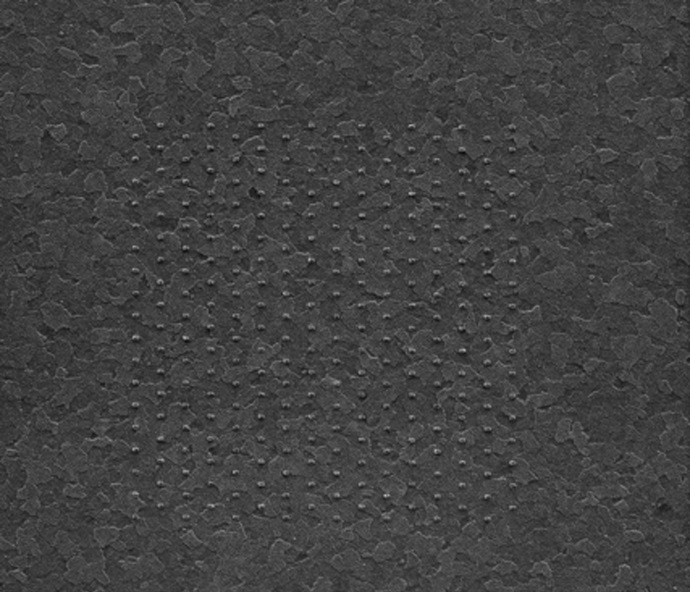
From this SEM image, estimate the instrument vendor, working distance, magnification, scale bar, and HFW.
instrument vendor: FEI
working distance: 9.9 mm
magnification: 17.803 K X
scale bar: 4000 nm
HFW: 8.38 µm